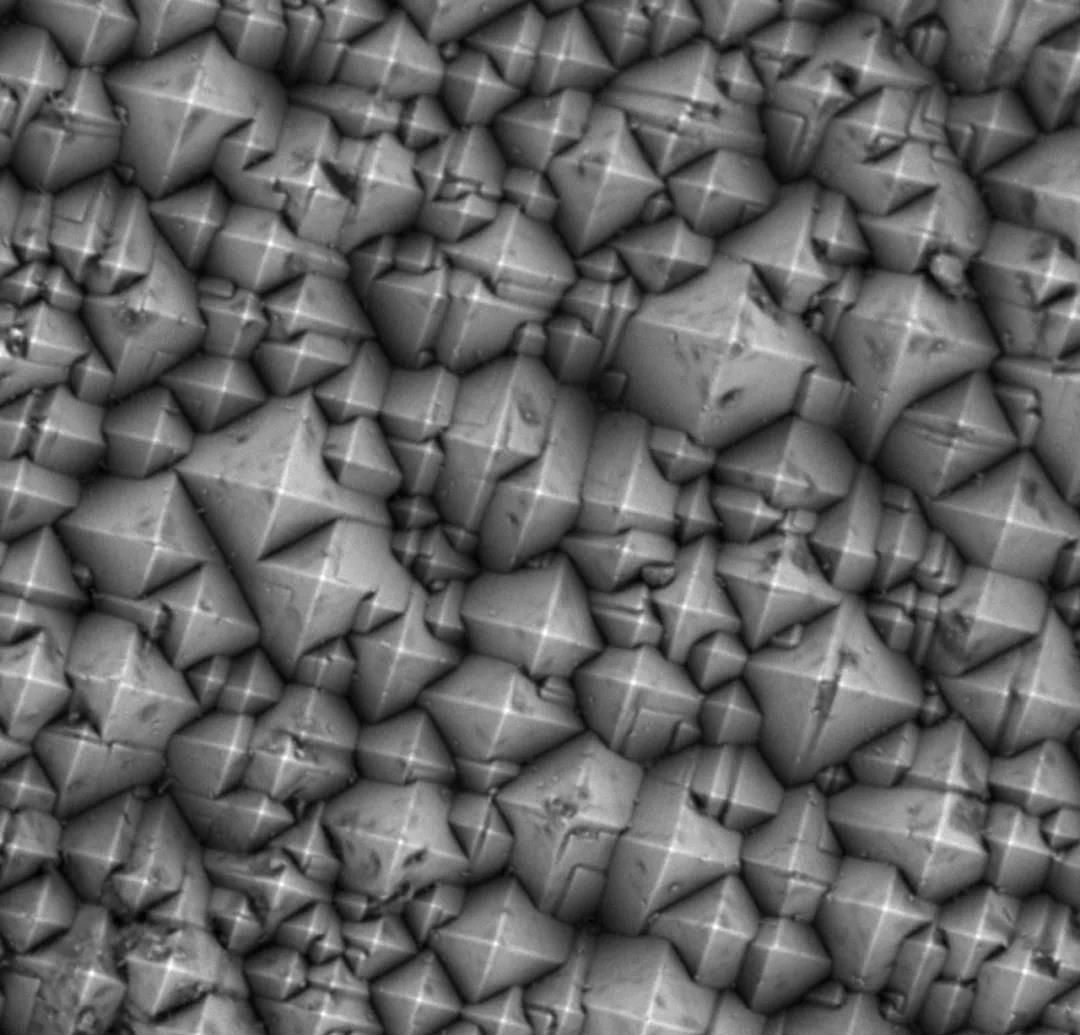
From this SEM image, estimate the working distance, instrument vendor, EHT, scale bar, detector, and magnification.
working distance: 6.1 mm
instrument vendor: other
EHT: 15 kV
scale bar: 5000 nm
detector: BSE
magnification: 10 K X